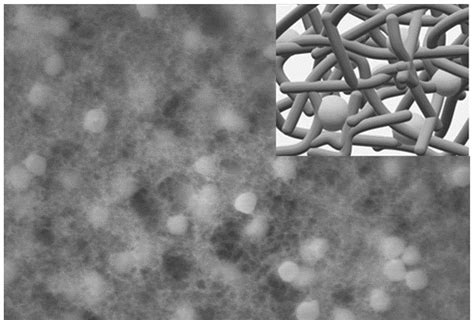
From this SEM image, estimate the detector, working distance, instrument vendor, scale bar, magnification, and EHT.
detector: SE(UL)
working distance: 9.4 mm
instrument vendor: Hitachi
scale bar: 1000 nm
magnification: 50 K X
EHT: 10 kV